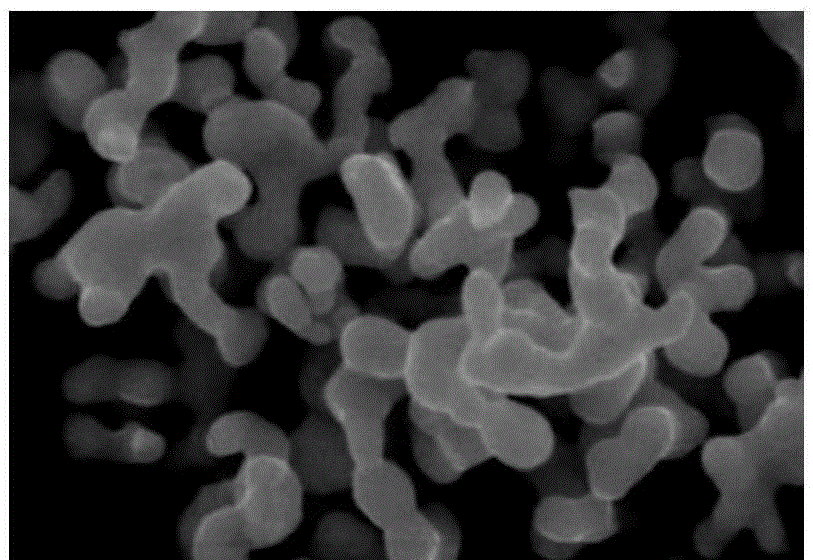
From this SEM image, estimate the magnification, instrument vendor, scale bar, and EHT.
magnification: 10 K X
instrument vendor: other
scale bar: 1000 nm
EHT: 25 kV